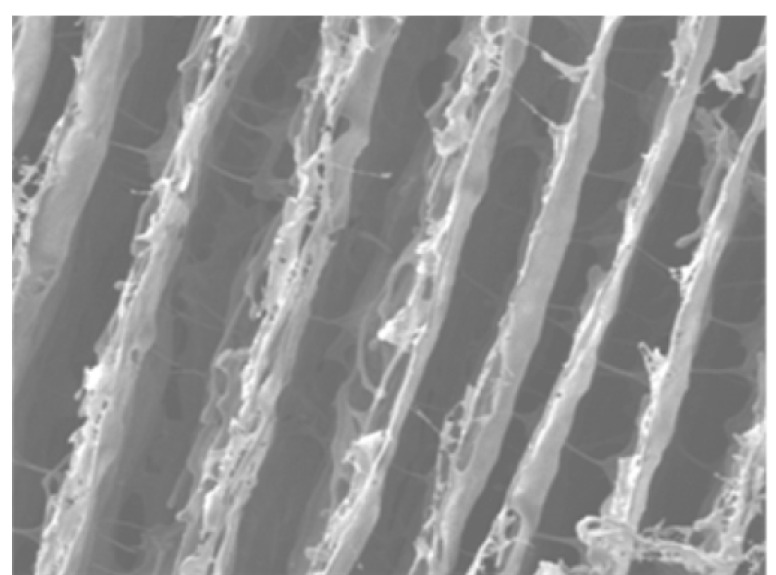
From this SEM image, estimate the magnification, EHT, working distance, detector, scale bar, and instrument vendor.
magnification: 0.6 K X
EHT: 5 kV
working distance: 8 mm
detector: SEI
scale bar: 10000 nm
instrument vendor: JEOL